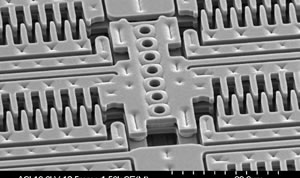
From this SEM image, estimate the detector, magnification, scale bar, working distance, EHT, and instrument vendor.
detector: SE(M)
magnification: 1.5 K X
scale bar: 30000 nm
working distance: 12.5 mm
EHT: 10 kV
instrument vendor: Hitachi